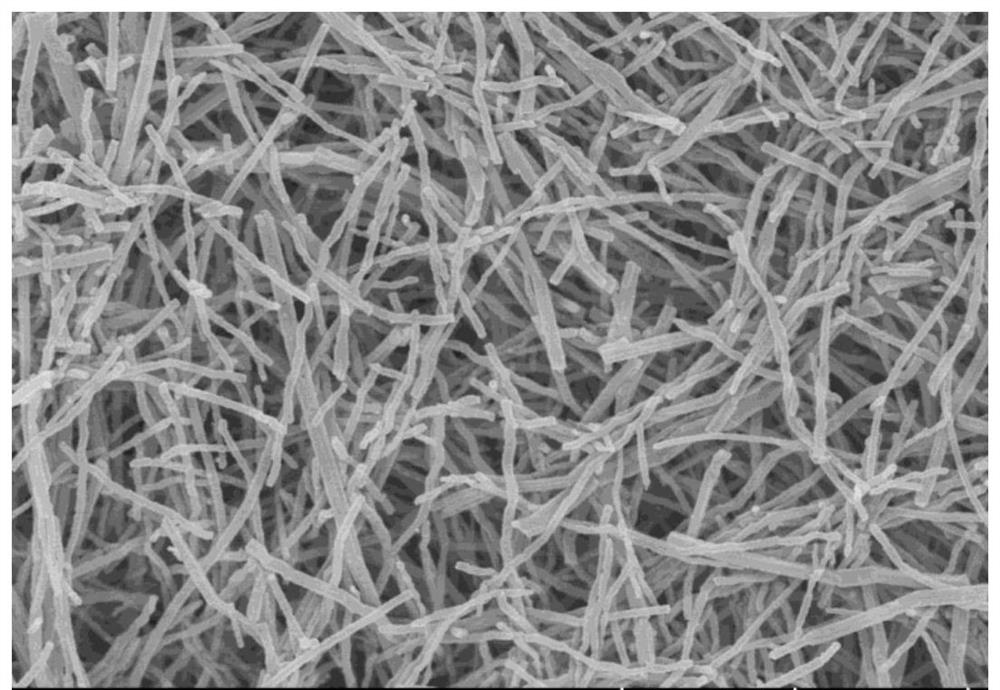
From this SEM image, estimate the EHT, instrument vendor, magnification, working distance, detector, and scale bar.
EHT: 10 kV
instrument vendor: Hitachi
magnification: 9 K X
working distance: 7.3 mm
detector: SE(U)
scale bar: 5000 nm